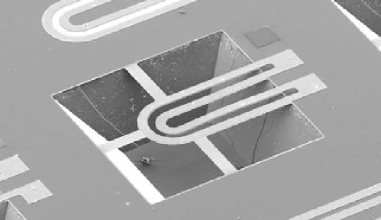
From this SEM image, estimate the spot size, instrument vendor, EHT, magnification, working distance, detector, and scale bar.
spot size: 5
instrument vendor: FEI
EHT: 10 kV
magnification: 0.2 K X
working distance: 19.4 mm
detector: SE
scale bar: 200000 nm